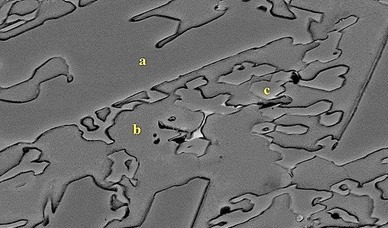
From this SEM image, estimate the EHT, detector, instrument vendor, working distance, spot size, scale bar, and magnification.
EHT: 20 kV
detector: BSE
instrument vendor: FEI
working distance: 10.2 mm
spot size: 4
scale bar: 5000 nm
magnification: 10 K X